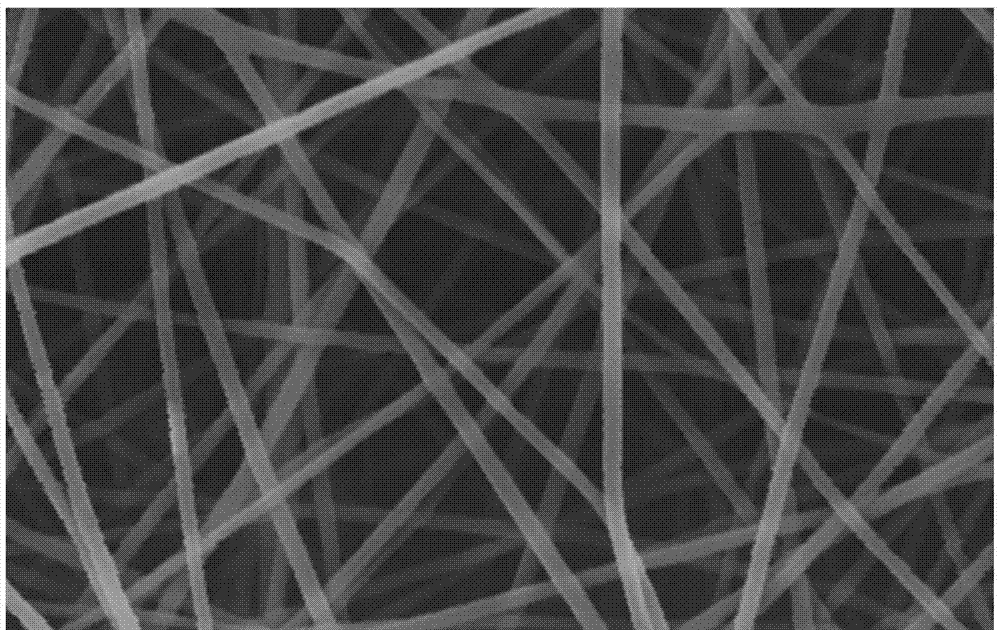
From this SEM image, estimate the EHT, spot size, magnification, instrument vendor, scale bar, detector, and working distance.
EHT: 15 kV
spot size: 4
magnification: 10 K X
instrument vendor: FEI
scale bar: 5000 nm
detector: SE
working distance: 18 mm